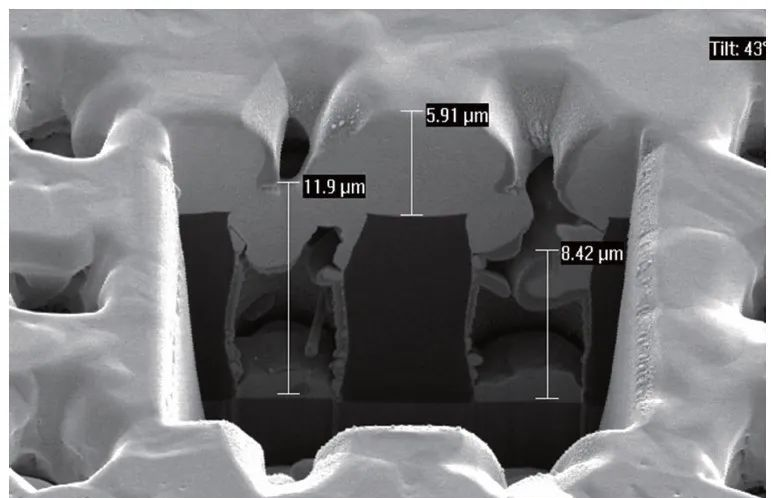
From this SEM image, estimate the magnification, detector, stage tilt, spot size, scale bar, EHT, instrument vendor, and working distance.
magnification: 4 K X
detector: SE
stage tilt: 43°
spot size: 3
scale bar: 5000 nm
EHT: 5 kV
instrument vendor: FEI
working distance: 5.2 mm